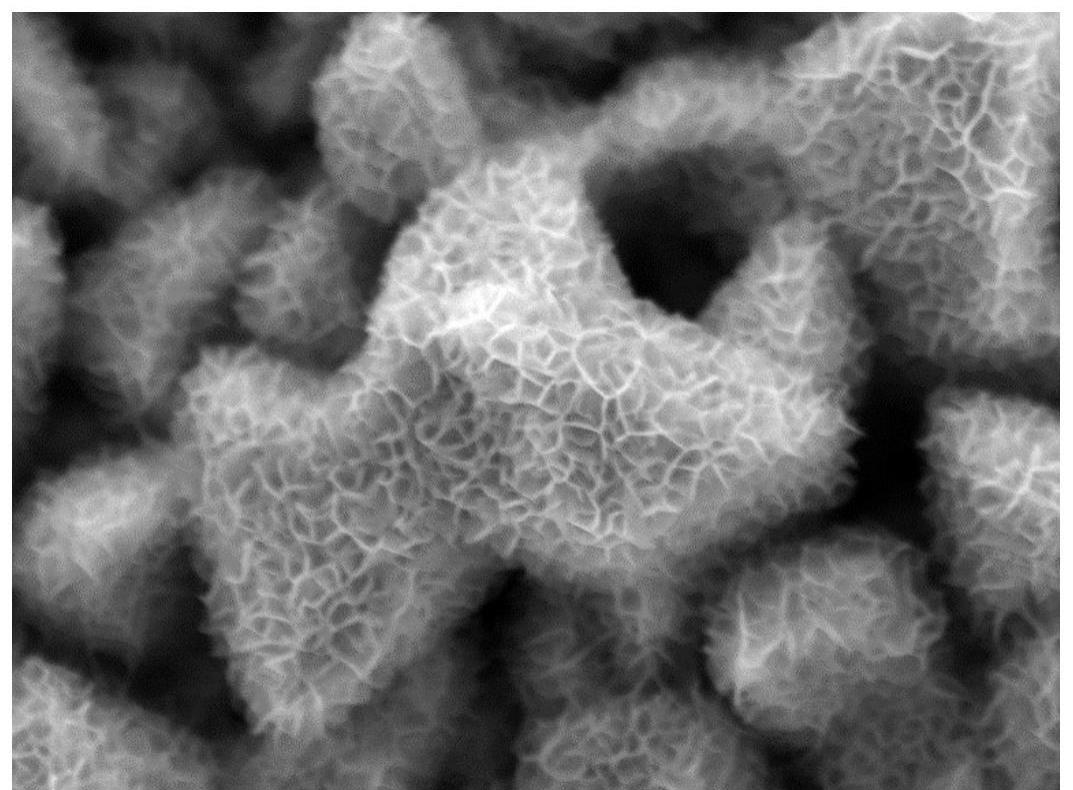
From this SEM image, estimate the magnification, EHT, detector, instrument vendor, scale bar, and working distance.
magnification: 15 K X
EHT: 10 kV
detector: LED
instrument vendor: JEOL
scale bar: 1000 nm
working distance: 7.4 mm